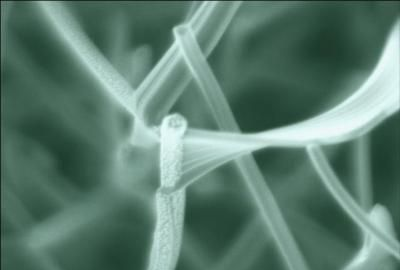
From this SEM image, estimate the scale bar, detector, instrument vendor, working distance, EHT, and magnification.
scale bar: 500 nm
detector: SE(U)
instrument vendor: Hitachi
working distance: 3 mm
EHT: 1 kV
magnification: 80 K X